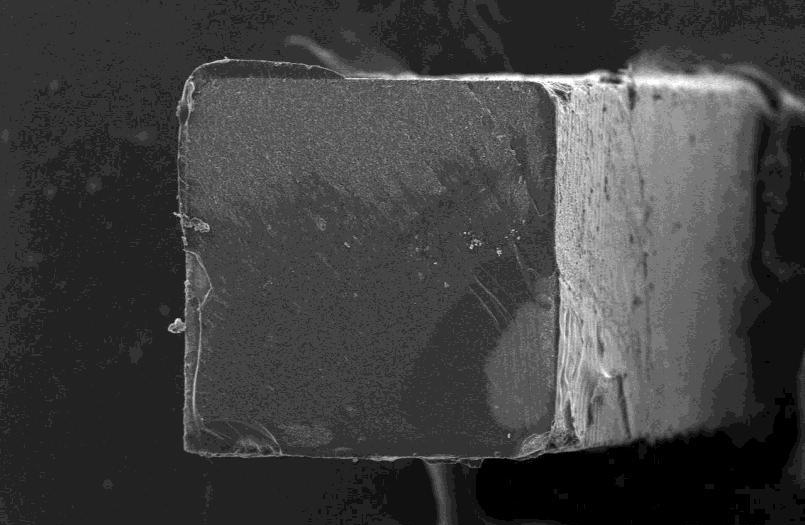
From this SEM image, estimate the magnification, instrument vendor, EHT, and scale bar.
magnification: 0.06 K X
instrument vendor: JEOL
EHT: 15 kV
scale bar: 200000 nm